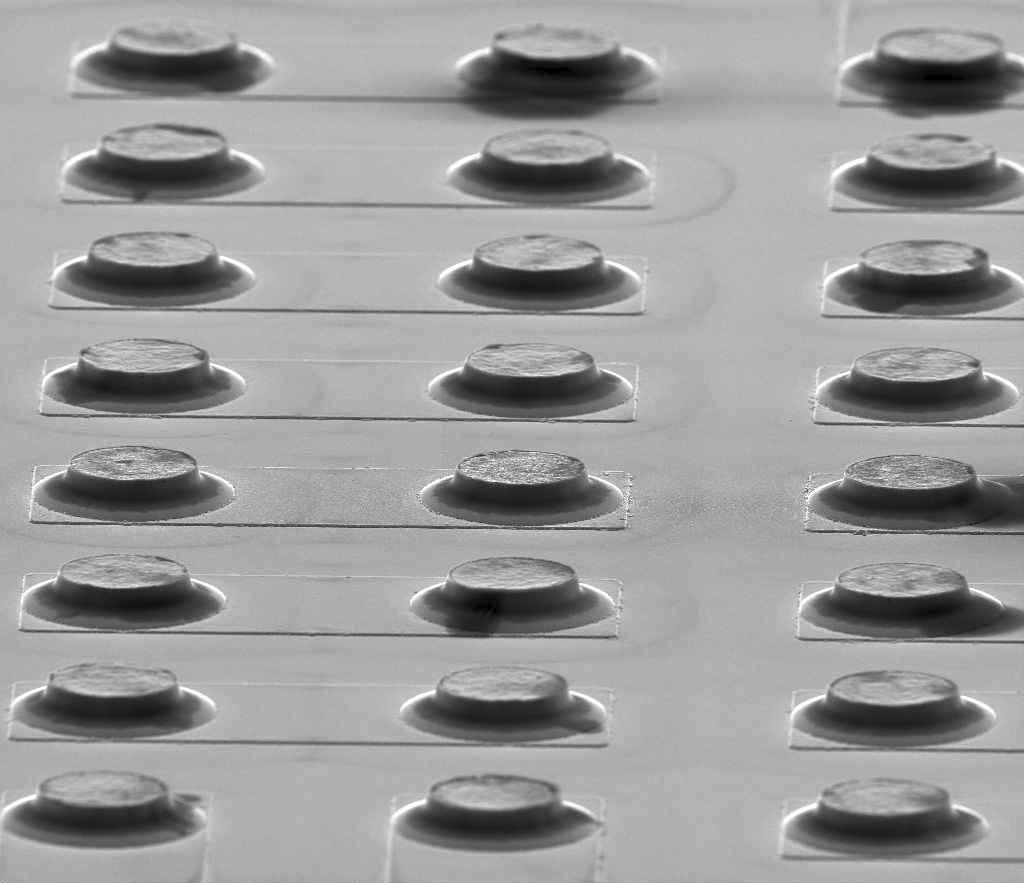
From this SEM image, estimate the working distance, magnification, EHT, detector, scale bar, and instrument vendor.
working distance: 15.1 mm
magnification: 1.5 K X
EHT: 10 kV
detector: SE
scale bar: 100000 nm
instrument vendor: FEI